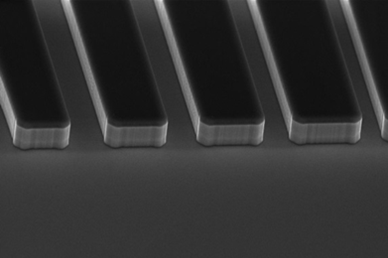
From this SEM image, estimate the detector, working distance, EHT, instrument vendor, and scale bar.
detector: InLens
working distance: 4.4 mm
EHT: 10 kV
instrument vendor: Zeiss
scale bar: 3000 nm